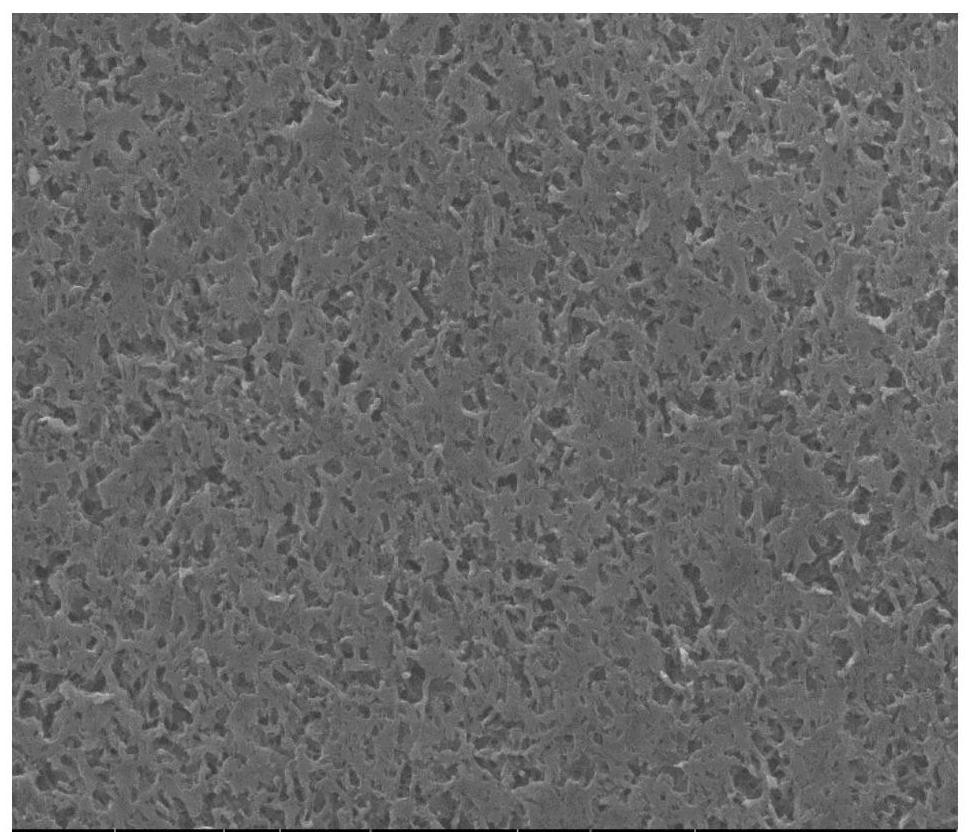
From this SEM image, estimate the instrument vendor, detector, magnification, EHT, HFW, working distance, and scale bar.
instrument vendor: FEI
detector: ETD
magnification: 2 K X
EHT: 20 kV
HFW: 67.6 µm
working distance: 10.2 mm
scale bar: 10000 nm